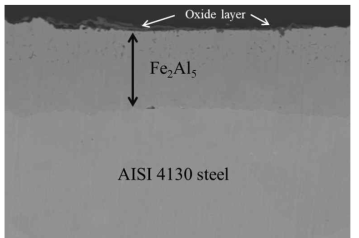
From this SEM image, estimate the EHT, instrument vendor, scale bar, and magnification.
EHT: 20 kV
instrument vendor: JEOL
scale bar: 10000 nm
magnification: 1 K X